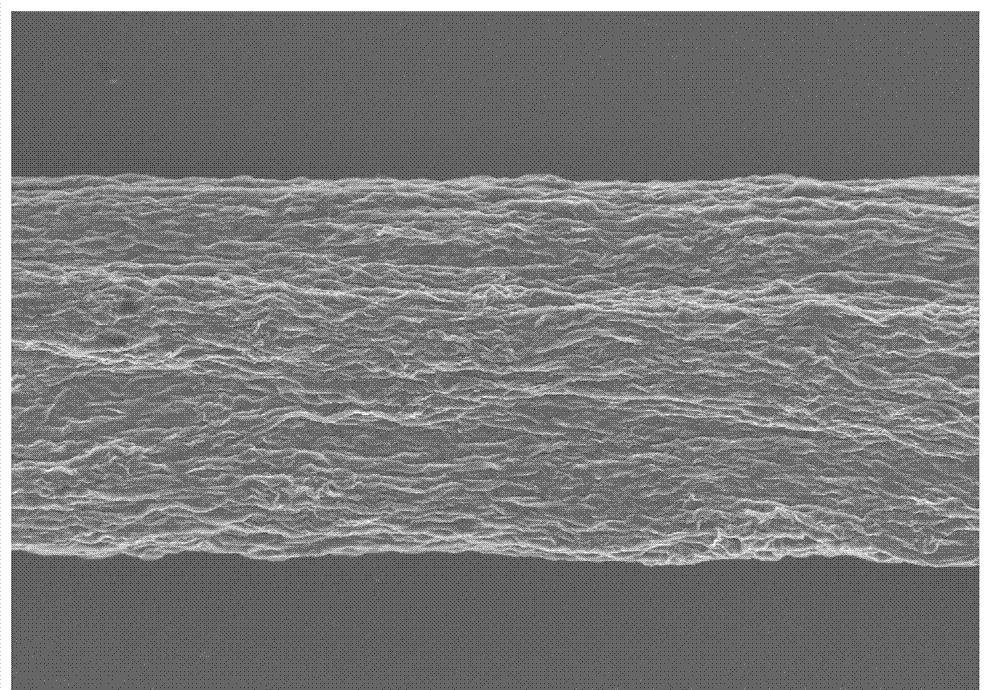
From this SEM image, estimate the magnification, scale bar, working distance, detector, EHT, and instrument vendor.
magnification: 0.5 K X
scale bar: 100000 nm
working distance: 9.6 mm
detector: SE(U)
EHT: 10 kV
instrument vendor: Hitachi